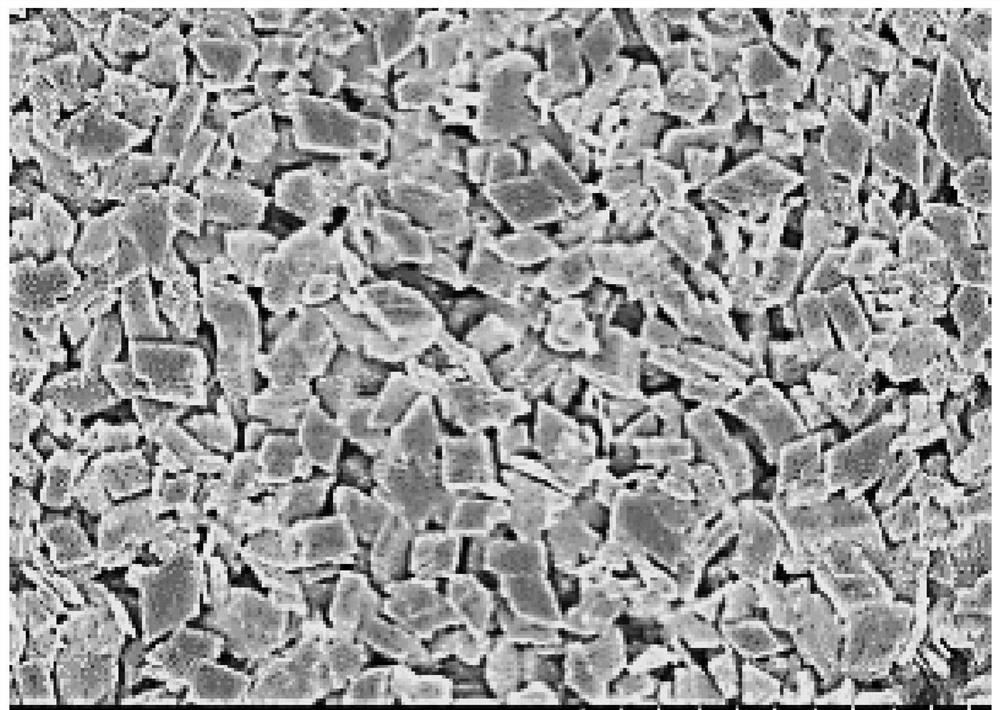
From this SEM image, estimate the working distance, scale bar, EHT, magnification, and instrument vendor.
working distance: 13.2 mm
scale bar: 5000 nm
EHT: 5 kV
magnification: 10 K X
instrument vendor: Hitachi